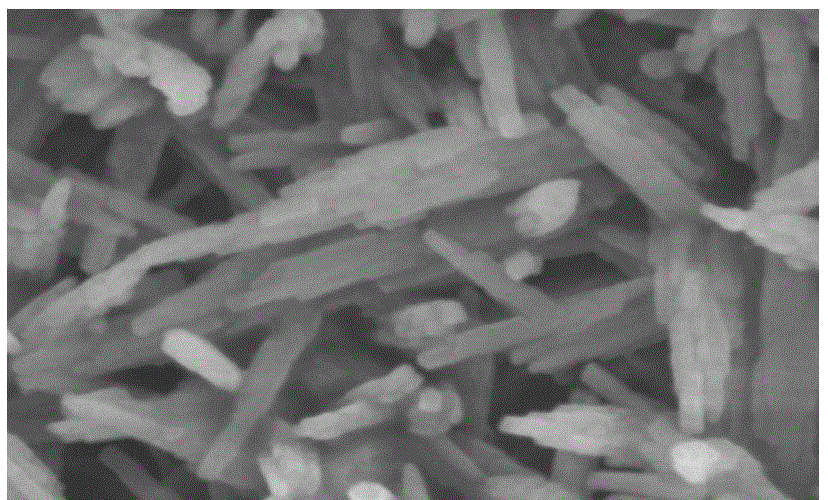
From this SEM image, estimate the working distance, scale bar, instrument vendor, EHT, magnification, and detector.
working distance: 9.6 mm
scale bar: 100 nm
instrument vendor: JEOL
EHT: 5 kV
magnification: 50 K X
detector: SEI